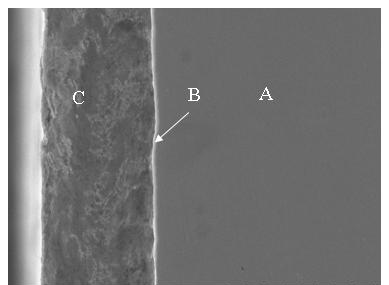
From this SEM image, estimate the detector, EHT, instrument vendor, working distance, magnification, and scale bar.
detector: SE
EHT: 20 kV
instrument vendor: Hitachi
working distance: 10.8 mm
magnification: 1 K X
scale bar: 50000 nm